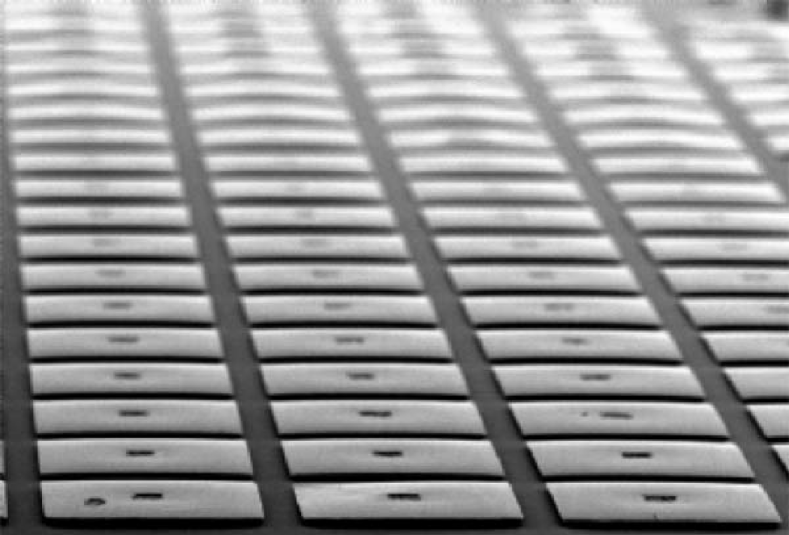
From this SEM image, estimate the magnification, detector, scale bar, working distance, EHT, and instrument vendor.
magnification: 0.07 K X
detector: SE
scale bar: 500000 nm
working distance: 12.3 mm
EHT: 25 kV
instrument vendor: Hitachi